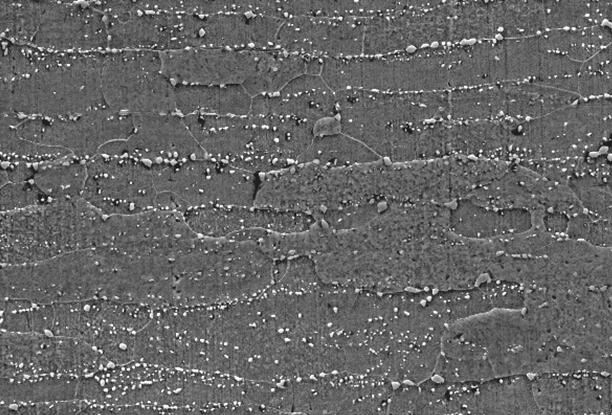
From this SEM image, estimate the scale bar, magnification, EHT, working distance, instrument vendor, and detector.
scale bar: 20000 nm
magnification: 5 K X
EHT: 20 kV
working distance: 8.9 mm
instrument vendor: FEI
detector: ETD